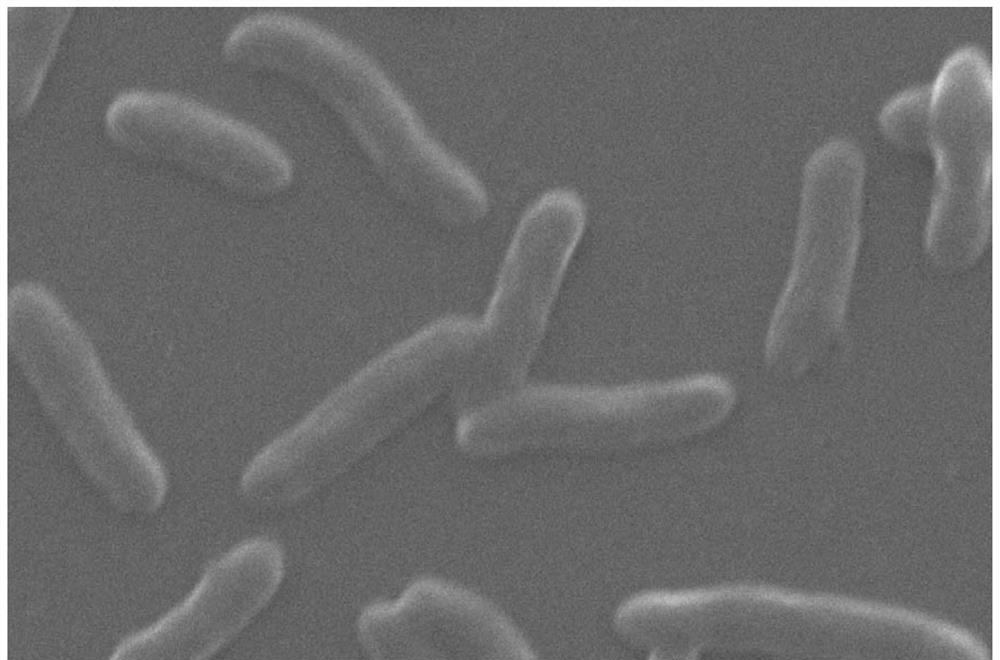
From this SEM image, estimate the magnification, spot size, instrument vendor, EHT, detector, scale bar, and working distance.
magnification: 65 K X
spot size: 3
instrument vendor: Thermo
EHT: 30 kV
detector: ETD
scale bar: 2000 nm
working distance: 13.8 mm